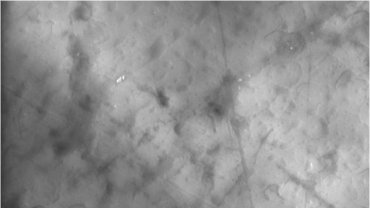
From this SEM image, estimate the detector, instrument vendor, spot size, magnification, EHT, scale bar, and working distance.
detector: GSE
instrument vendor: FEI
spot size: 5.1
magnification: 2 K X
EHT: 20 kV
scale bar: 10000 nm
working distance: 10.1 mm